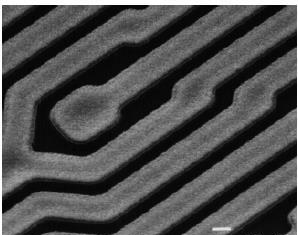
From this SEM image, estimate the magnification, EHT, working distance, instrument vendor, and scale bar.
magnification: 5 K X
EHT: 25 kV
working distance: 8 mm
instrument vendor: JEOL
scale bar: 1000 nm